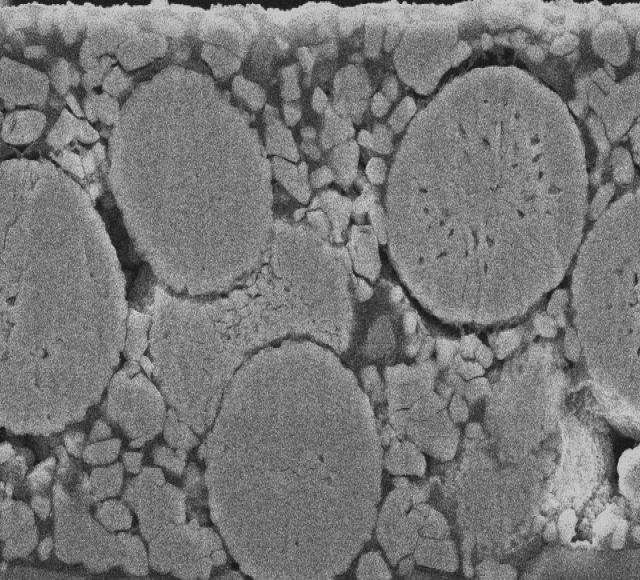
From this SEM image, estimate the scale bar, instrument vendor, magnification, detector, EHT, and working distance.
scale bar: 5000 nm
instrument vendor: JEOL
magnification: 3 K X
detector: SEI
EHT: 15 kV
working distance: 8 mm